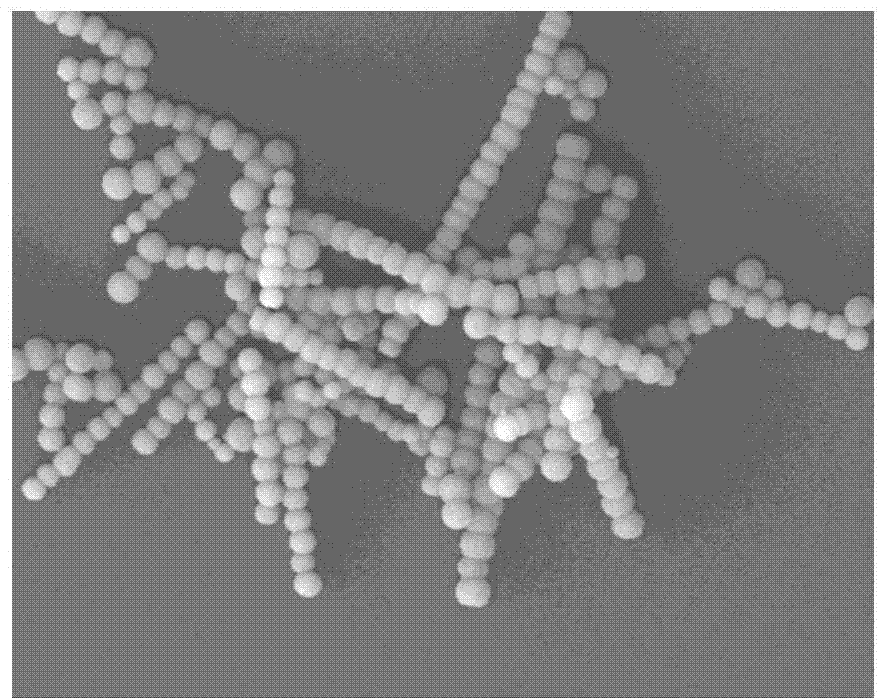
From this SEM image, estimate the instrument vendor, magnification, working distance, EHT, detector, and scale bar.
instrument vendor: JEOL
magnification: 5 K X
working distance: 8 mm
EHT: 20 kV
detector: LEI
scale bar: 1000 nm